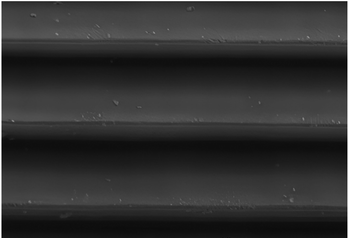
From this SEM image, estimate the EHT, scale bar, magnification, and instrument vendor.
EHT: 15 kV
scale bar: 10000 nm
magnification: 2.4 K X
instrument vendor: JEOL